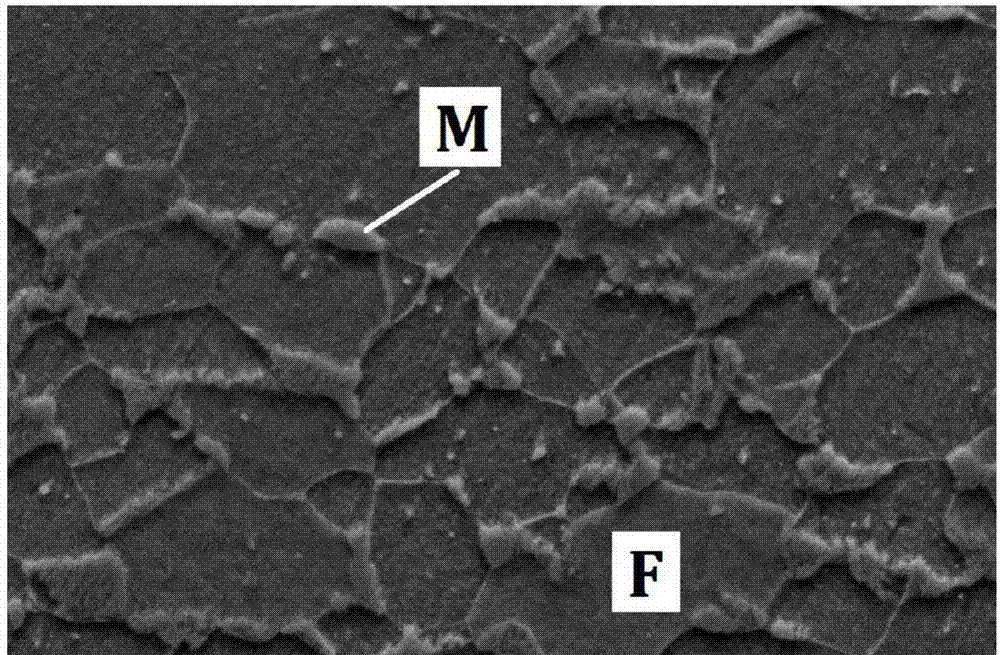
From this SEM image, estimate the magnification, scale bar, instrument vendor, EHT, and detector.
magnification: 3 K X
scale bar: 5000 nm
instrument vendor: JEOL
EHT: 20 kV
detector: SEI